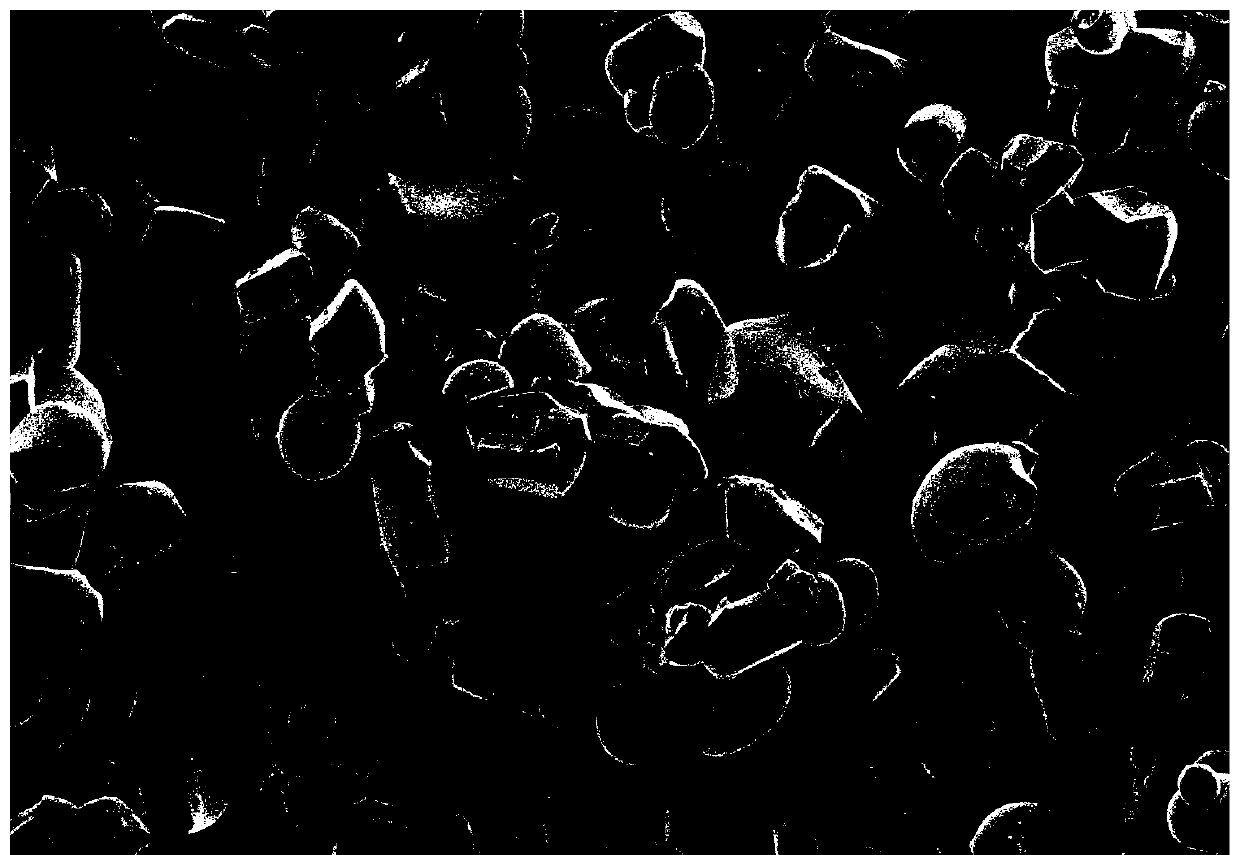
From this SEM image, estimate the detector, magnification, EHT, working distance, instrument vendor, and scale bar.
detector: SE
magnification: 2 K X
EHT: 5 kV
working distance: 4.8 mm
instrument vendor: Hitachi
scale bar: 20000 nm